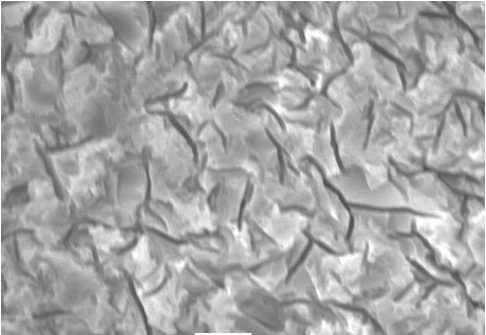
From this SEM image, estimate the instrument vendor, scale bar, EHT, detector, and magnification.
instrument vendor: JEOL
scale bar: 5000 nm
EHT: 20 kV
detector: SEI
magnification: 3 K X